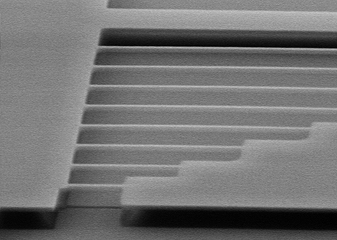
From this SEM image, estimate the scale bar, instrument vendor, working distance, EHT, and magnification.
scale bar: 2000 nm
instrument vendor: Hitachi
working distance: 5 mm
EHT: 5 kV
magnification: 20 K X